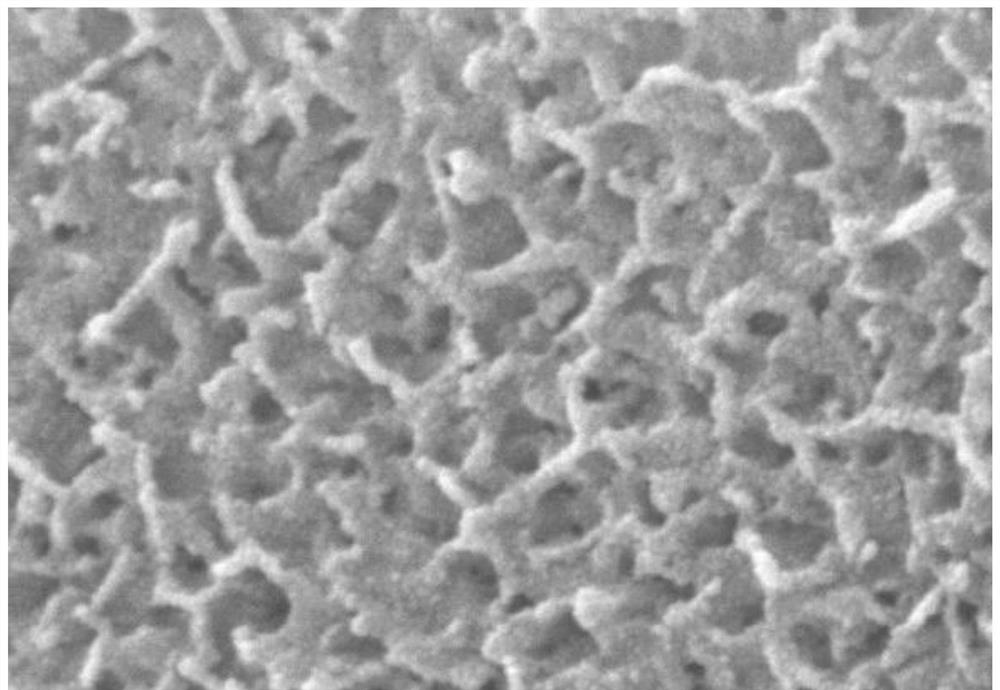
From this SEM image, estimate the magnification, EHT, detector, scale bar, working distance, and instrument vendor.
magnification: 30 K X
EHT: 15 kV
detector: SE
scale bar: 1000 nm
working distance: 7.5 mm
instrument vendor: Hitachi